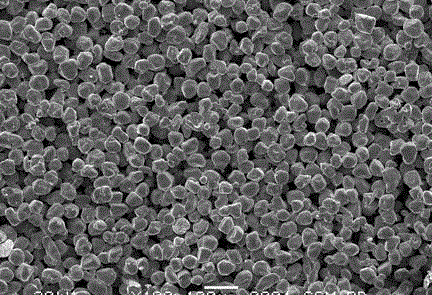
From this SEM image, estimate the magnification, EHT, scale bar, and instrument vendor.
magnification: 0.1 K X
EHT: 30 kV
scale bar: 100000 nm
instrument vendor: JEOL